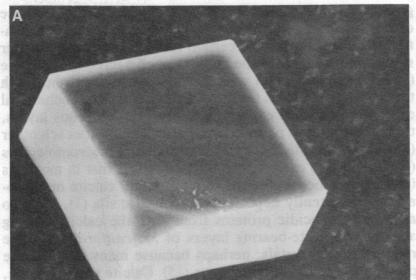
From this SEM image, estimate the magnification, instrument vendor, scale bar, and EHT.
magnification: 1.3 K X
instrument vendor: JEOL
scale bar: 10000 nm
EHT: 25 kV